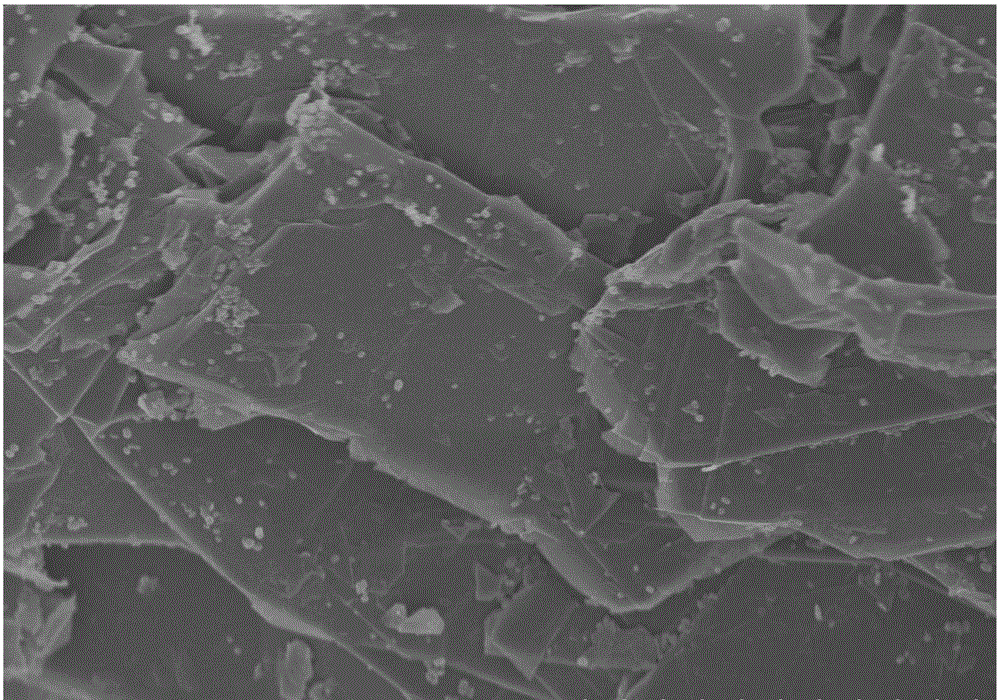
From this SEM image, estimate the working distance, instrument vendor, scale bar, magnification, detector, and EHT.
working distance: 8.6 mm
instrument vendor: Hitachi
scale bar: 10000 nm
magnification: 5 K X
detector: SE(M)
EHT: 5 kV